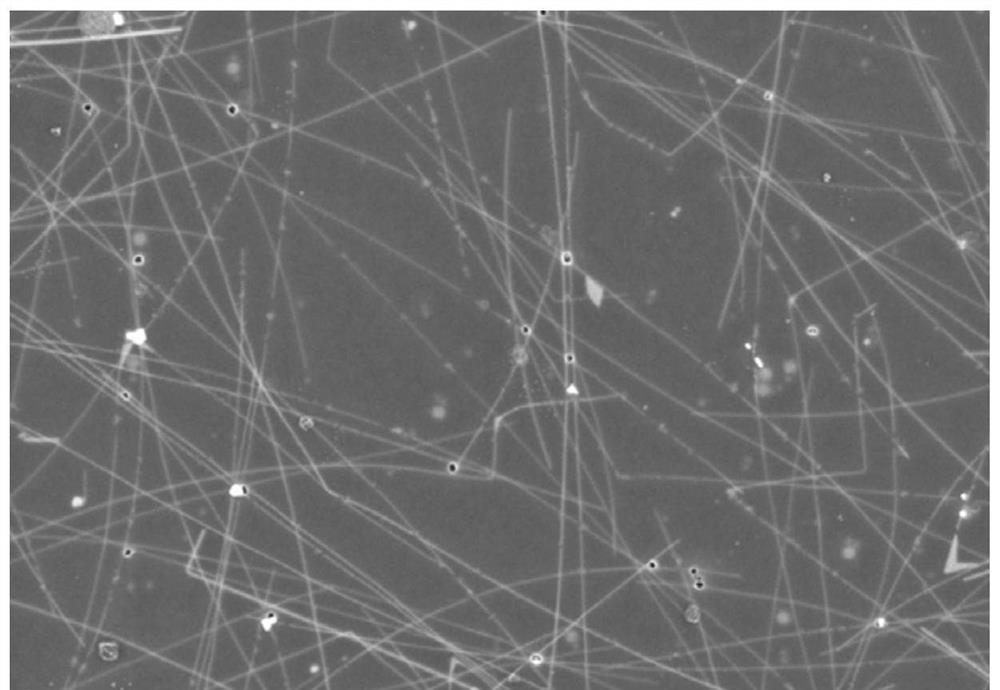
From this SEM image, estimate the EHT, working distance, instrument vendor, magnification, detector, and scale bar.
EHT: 5 kV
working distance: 4.9 mm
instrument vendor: Zeiss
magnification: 10 K X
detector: InLens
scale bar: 1000 nm